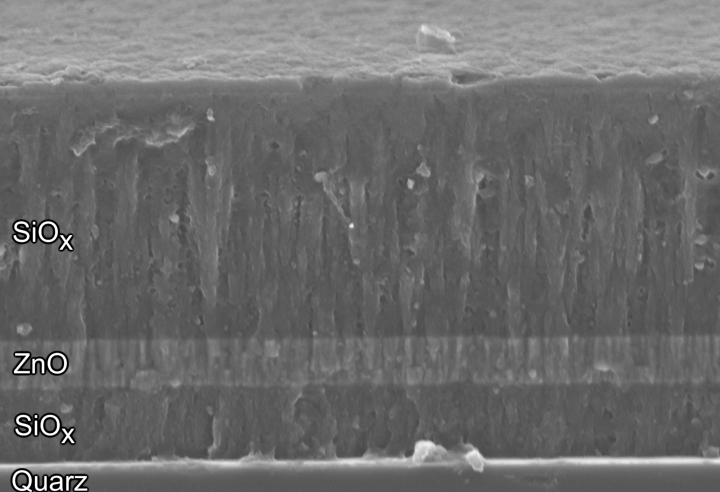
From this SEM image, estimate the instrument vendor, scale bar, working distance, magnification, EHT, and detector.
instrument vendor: Zeiss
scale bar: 1000 nm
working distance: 4 mm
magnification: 25 K X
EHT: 5 kV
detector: InLens